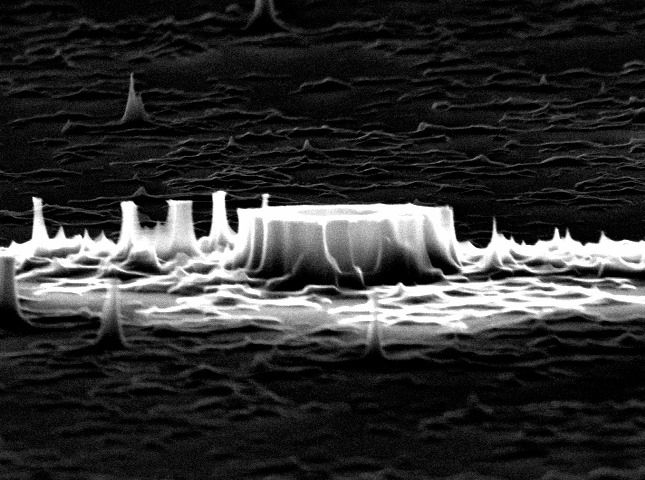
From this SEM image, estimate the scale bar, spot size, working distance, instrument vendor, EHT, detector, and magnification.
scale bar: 1000 nm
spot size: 3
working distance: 18.4 mm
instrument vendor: FEI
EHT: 30 kV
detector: SE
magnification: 24.564 K X